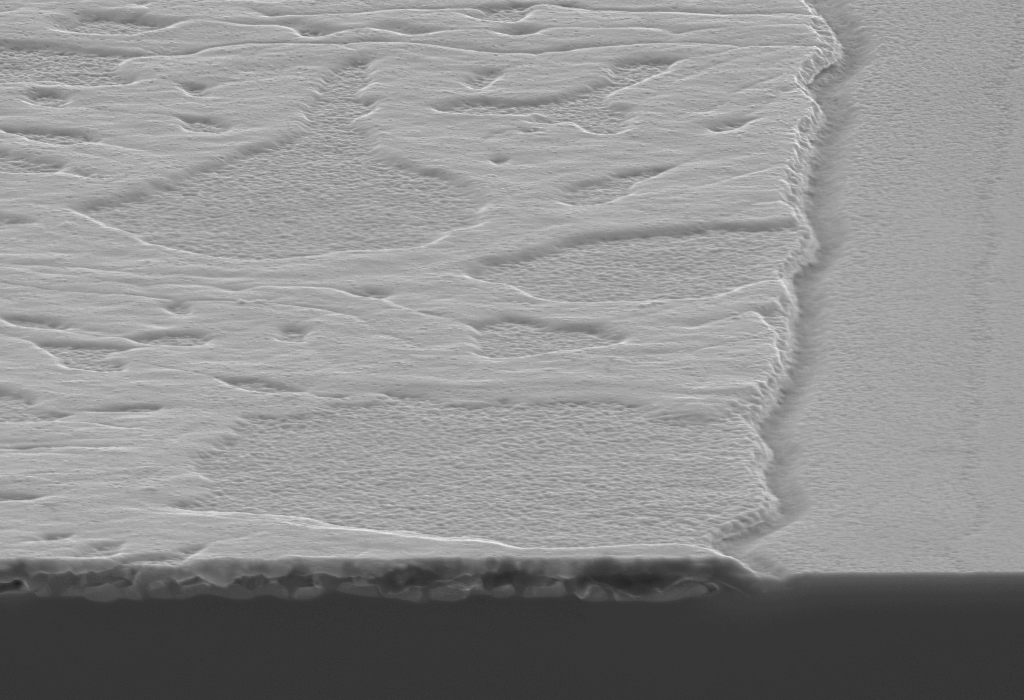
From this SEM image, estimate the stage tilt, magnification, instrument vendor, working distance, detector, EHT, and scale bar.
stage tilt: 10.2°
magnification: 50 K X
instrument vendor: Zeiss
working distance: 3.1 mm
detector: InLens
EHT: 7 kV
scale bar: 200 nm